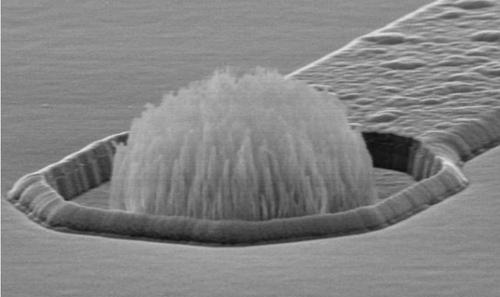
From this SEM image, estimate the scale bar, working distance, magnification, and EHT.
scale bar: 5000 nm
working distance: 17 mm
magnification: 3.7 K X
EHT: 15 kV